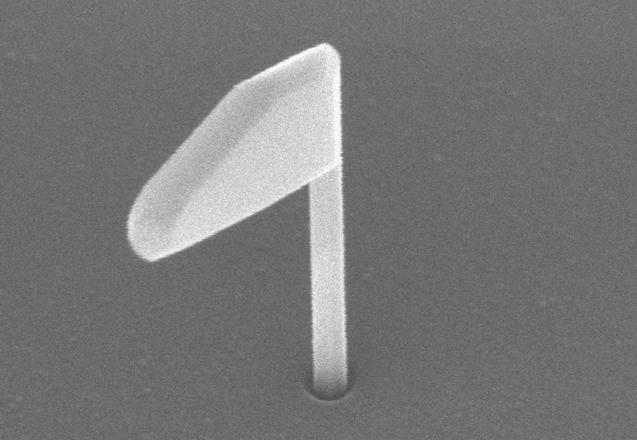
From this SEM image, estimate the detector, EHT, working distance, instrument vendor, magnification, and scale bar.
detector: SE(U)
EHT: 5 kV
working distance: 5.4 mm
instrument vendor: Hitachi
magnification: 130 K X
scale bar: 400 nm